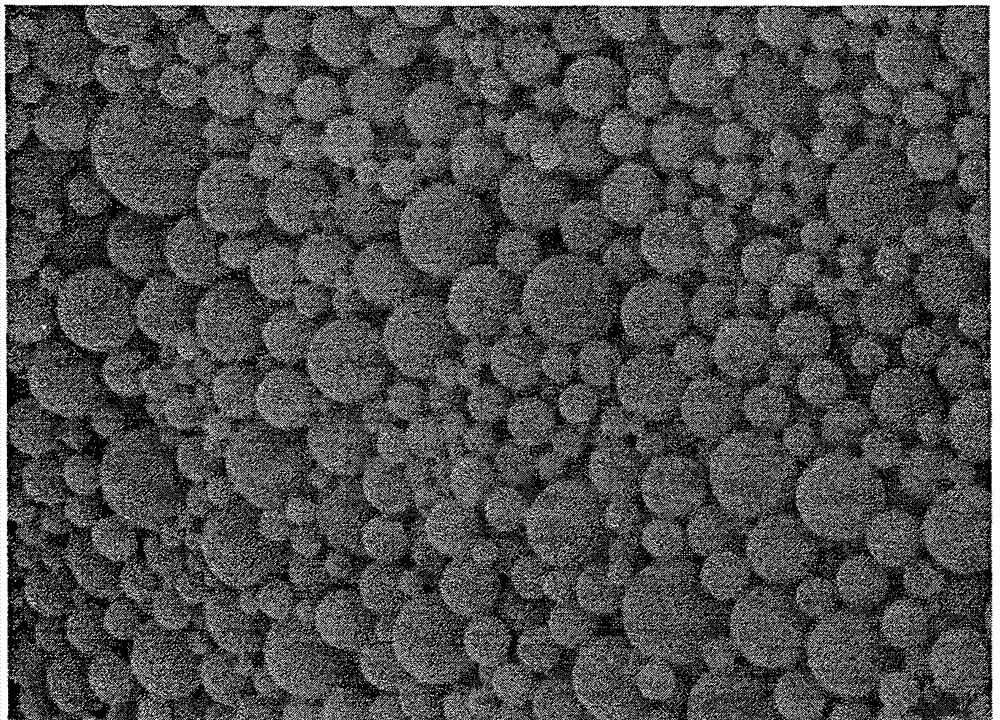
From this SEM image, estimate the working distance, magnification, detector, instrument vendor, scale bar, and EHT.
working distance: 17 mm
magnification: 15 K X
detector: SEI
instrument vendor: JEOL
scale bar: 1000 nm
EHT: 5 kV